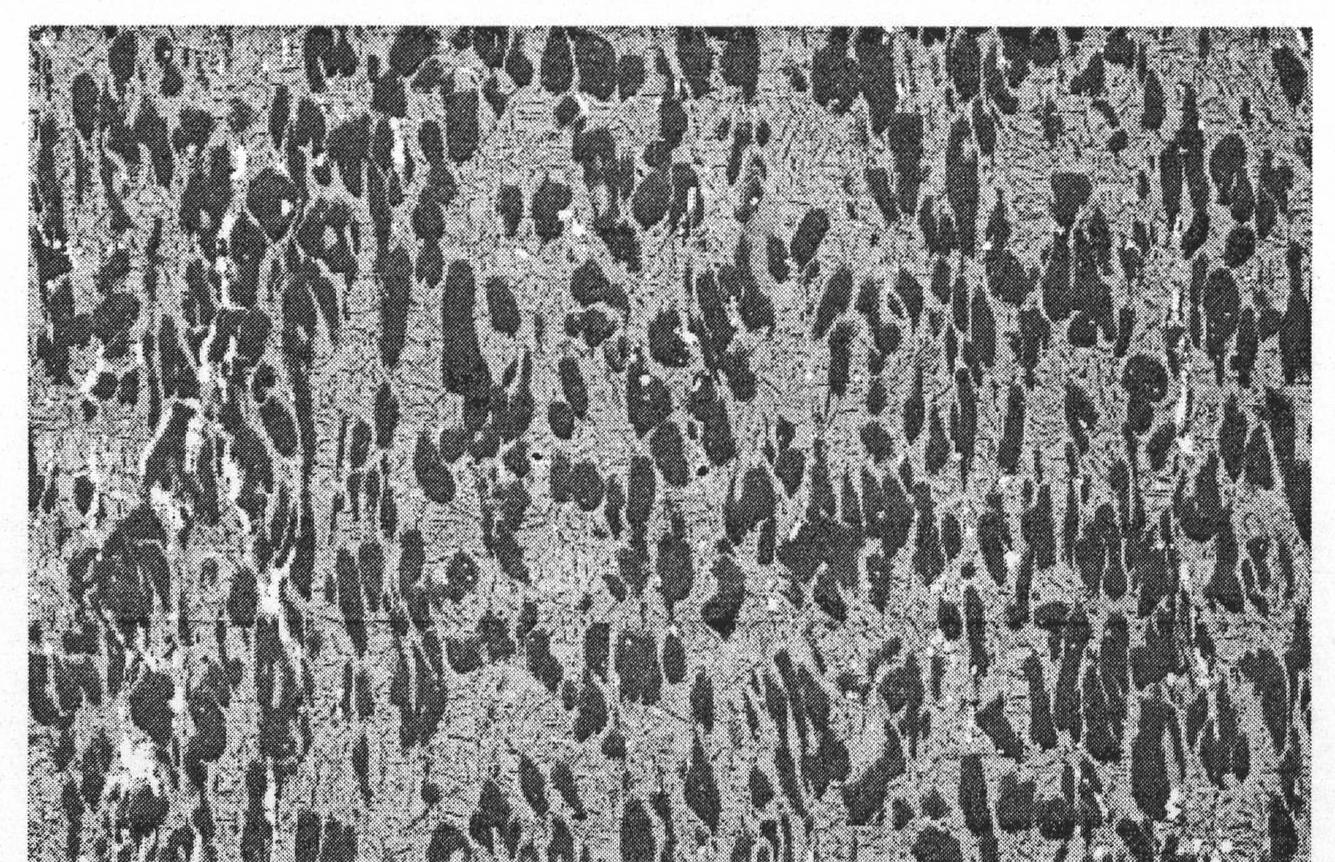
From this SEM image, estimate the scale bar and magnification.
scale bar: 100000 nm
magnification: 0.2 K X